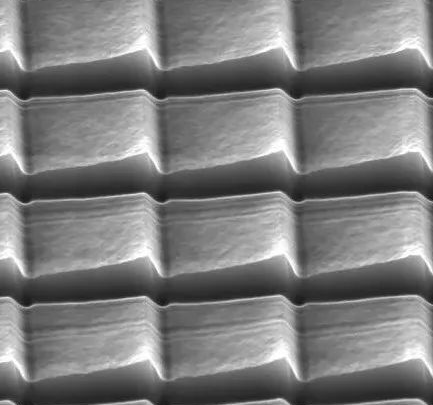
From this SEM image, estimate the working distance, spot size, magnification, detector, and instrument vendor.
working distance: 13.9 mm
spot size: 10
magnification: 0.8 K X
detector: ETD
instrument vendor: FEI